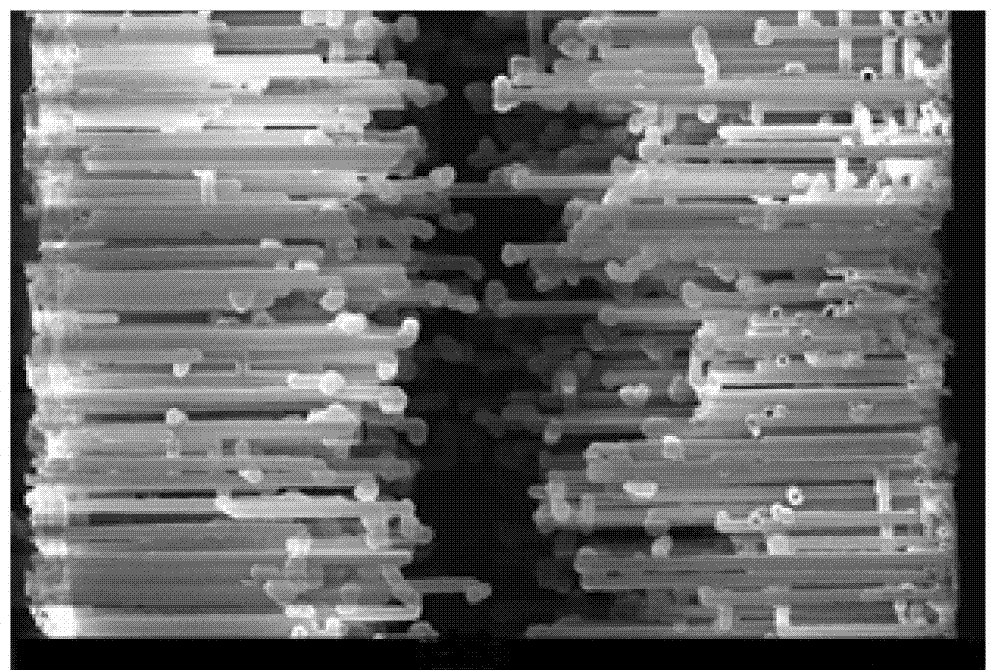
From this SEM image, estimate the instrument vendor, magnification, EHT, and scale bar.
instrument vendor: other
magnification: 0.95 K X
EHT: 15 kV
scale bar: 100000 nm